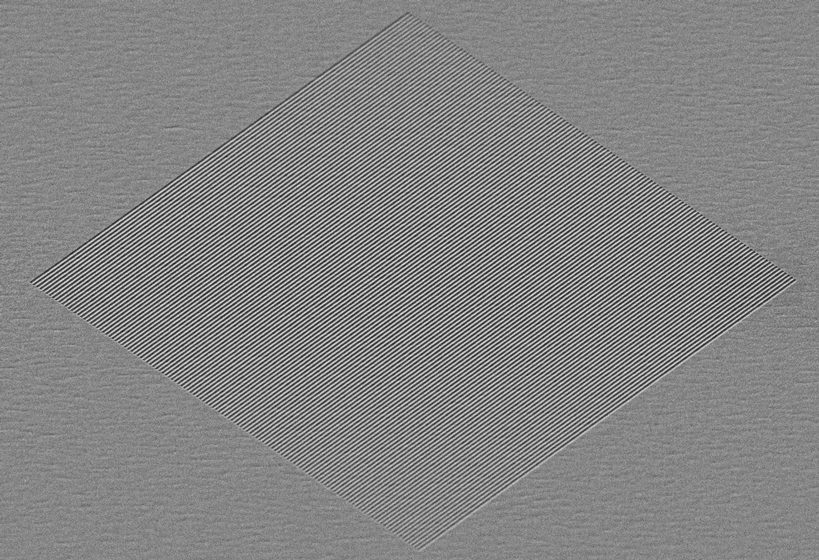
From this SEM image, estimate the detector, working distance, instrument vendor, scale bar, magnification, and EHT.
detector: SE2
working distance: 6.7 mm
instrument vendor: Zeiss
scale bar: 1000 nm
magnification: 3 K X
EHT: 5 kV